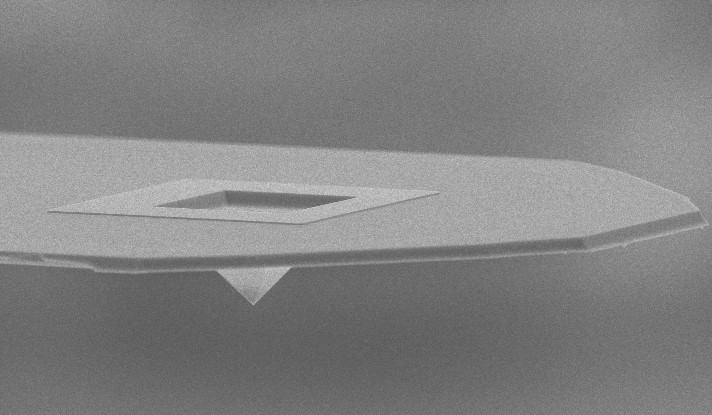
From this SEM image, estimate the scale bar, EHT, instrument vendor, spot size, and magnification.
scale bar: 20000 nm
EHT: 10 kV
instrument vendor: FEI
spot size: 3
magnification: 1.015 K X